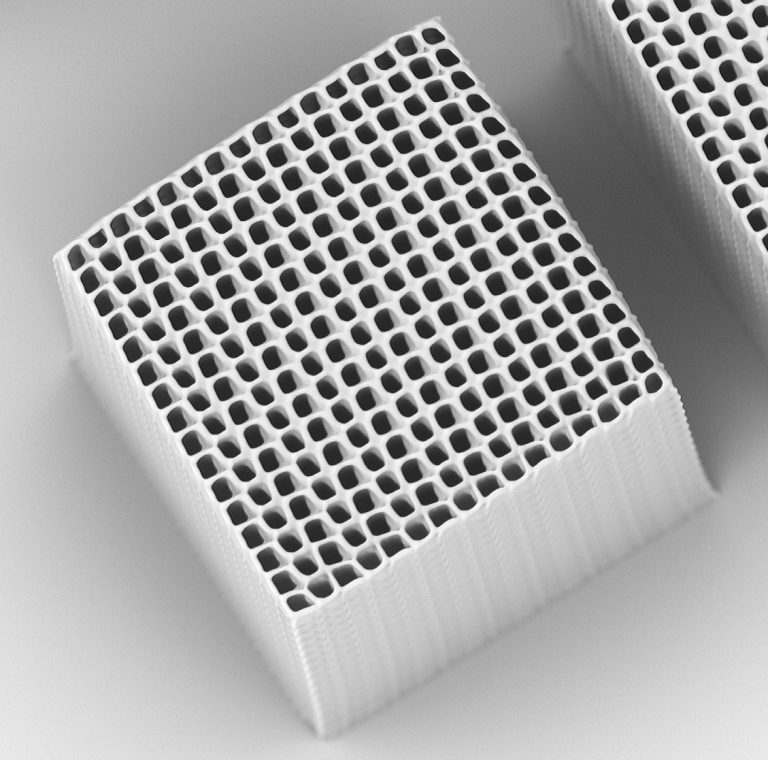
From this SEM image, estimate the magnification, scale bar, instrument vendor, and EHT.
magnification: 1.05 K X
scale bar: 80000 nm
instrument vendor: other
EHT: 15 kV